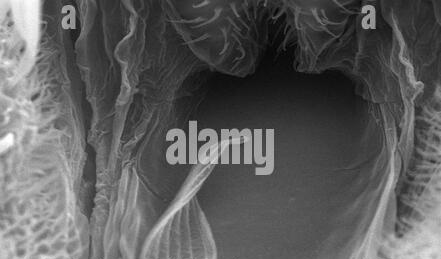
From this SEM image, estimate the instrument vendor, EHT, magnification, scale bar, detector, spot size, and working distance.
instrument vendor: FEI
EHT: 30 kV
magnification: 1.828 K X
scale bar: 20000 nm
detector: SE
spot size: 3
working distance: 6 mm